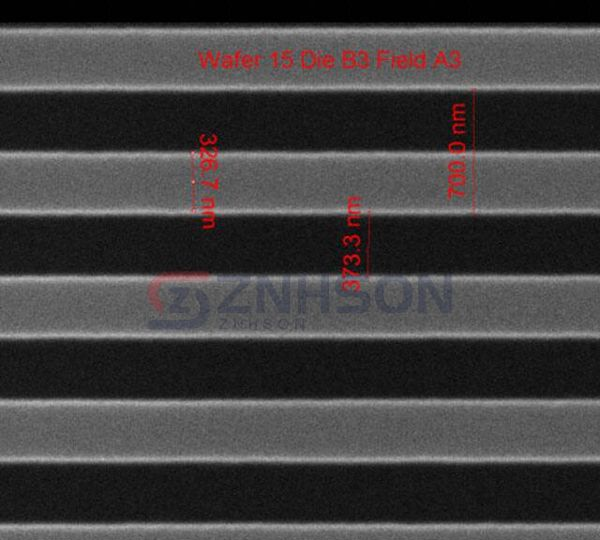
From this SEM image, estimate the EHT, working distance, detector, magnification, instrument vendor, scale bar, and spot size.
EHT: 10 kV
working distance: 10.2 mm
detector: ETD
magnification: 75.019 K X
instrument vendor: FEI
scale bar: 1000 nm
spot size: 1.5